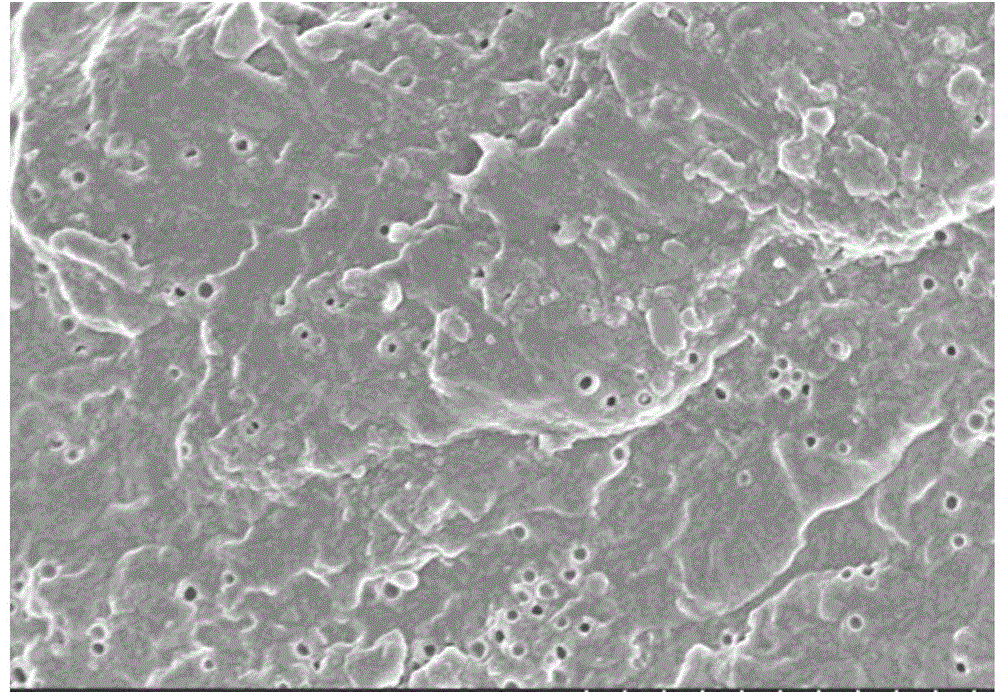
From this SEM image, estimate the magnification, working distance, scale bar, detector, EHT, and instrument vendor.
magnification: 10 K X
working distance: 6.4 mm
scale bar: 5000 nm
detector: SE(M)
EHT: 3 kV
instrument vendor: Hitachi